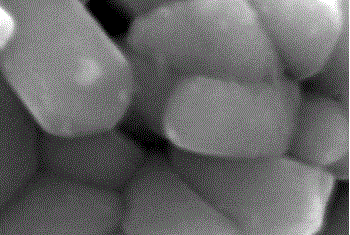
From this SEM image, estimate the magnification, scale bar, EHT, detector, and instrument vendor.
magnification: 30 K X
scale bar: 500 nm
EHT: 20 kV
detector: SEI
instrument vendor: JEOL